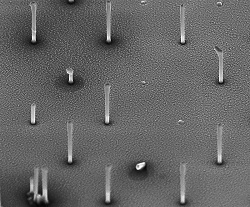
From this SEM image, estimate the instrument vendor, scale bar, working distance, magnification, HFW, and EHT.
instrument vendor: FEI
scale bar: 5000 nm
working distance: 4.2 mm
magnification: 15 K X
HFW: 17.1 µm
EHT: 5 kV